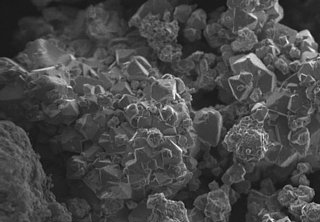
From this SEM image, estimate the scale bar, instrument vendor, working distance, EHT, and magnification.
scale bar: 5000 nm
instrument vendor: other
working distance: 3.7 mm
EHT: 2 kV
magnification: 2 K X